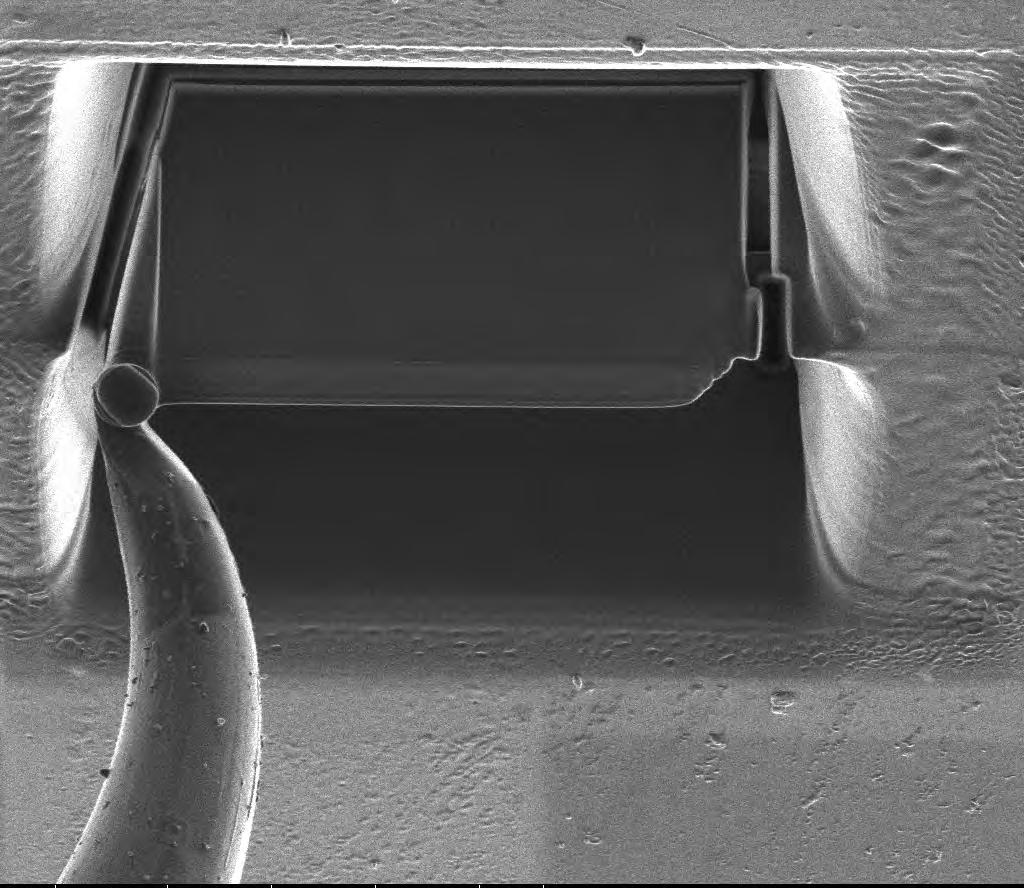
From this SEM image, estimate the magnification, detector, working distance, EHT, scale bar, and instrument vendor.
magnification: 5 K X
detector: ETD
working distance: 19 mm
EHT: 30 kV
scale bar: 10000 nm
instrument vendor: FEI